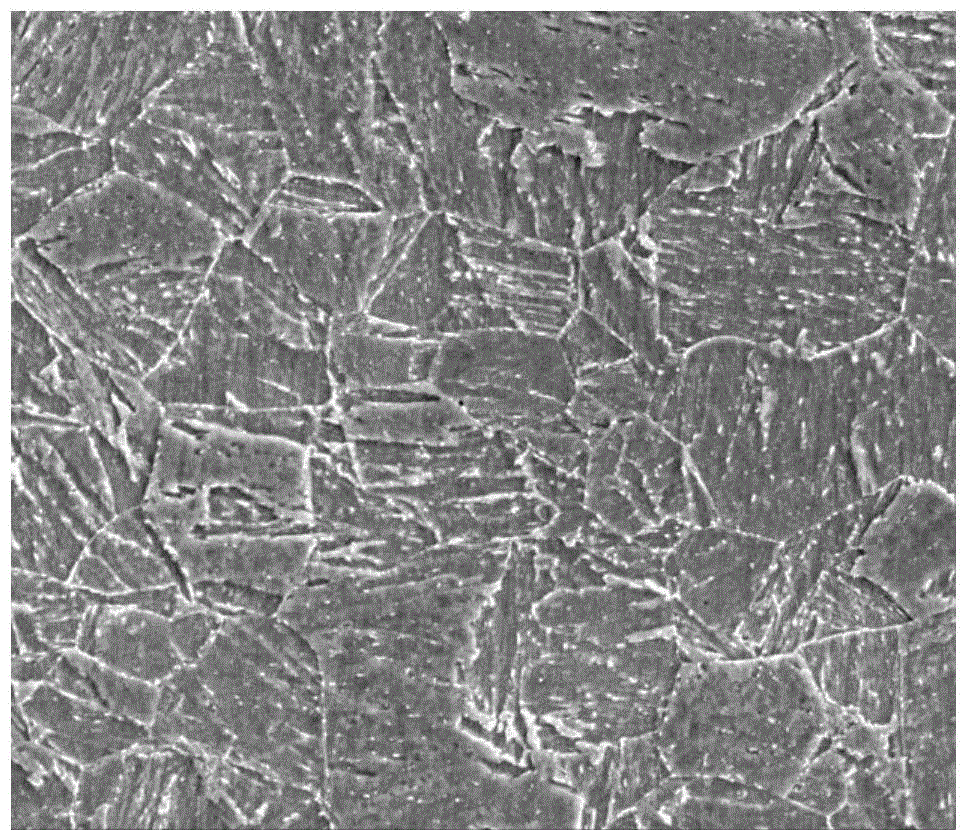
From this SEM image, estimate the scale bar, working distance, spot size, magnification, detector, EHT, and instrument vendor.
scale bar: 20000 nm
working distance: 11.9 mm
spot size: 4.5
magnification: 2 K X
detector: ETD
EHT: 20 kV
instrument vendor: FEI